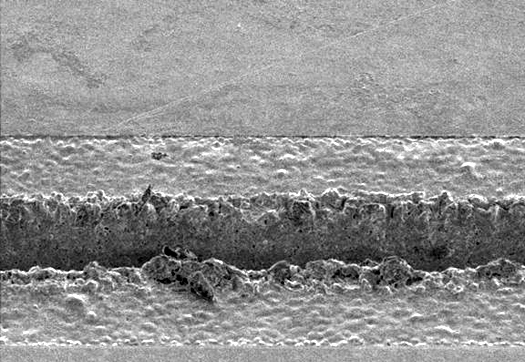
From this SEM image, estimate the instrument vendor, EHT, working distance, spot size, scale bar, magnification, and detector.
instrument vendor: FEI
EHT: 5 kV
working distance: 11.2 mm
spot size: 3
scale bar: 50000 nm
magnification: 0.35 K X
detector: SE